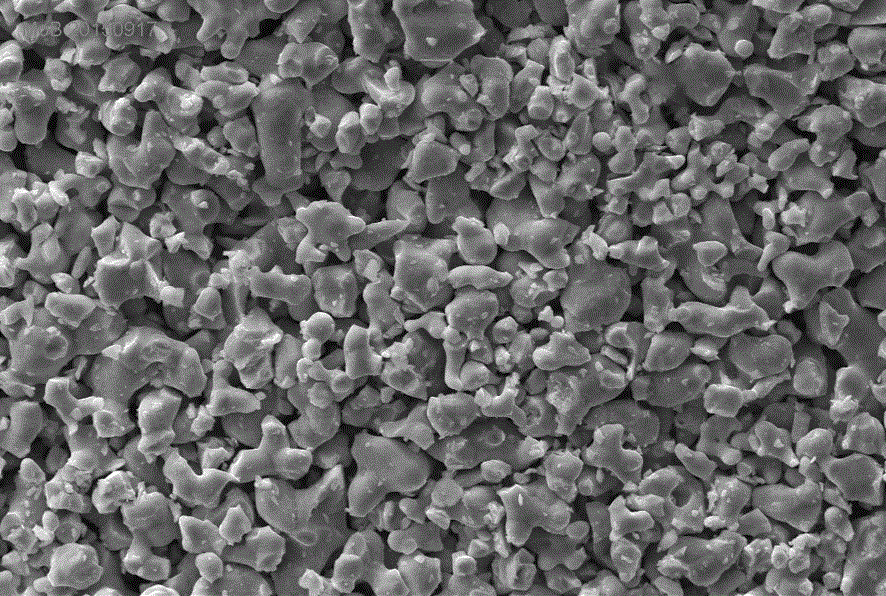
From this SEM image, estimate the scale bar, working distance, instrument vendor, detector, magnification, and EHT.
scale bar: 10000 nm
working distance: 10 mm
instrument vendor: Zeiss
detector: SE1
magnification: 1.5 K X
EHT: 20 kV